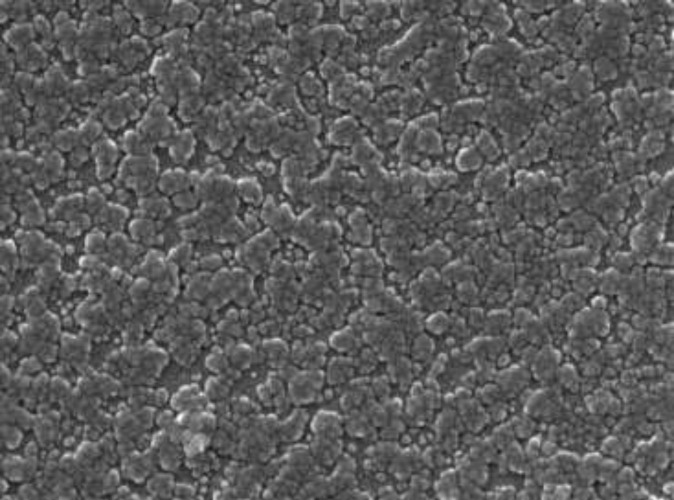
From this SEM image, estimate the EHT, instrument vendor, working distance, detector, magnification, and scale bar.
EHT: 20 kV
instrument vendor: Tescan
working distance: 9.99 mm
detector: InBeam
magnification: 150 K X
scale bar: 500 nm